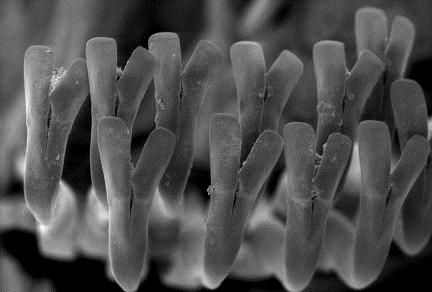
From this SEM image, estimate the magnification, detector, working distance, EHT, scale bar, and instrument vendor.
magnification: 0.17 K X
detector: SE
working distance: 3 mm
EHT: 15 kV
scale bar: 100000 nm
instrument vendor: other